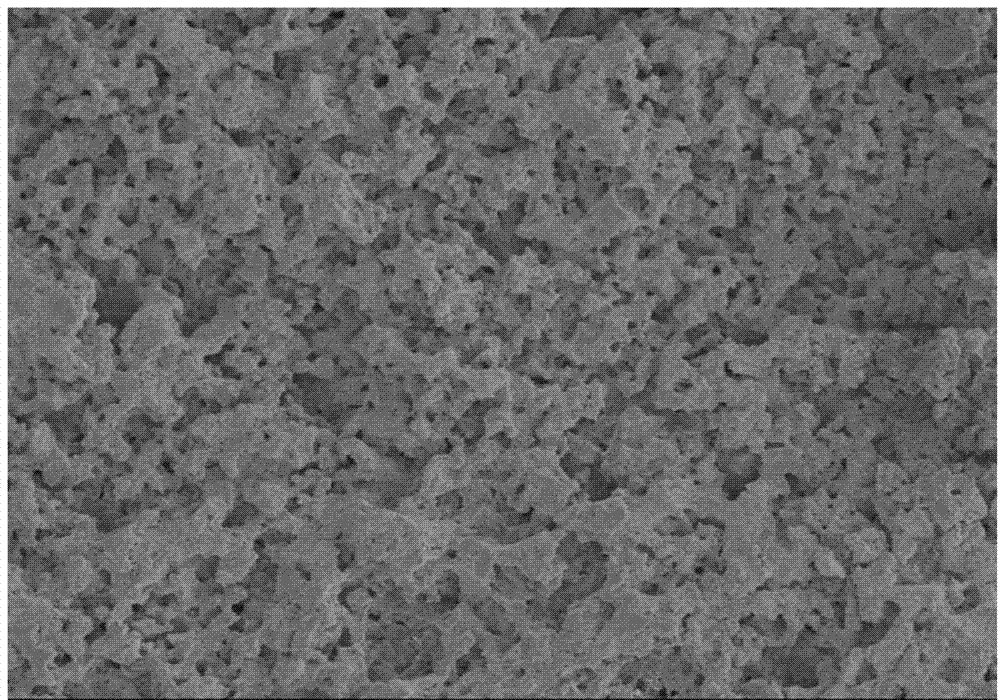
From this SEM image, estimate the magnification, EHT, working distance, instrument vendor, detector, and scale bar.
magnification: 1 K X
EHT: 5 kV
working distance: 10.8 mm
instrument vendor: Hitachi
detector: SE(M)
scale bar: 50000 nm